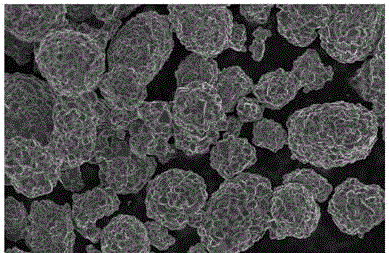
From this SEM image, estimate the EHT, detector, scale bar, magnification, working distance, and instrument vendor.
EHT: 5 kV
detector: InLens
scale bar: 2000 nm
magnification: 2 K X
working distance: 7.5 mm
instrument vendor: Zeiss